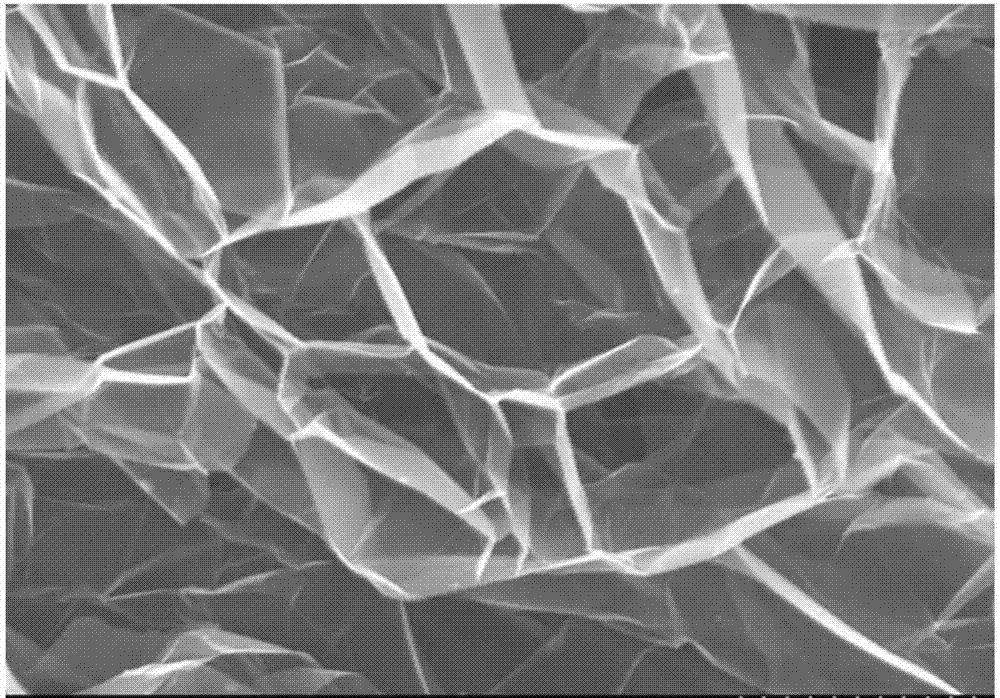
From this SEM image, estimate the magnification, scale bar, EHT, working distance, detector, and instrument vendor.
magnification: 3 K X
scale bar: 10000 nm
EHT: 10 kV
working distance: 12.8 mm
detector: SE(M)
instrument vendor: Hitachi